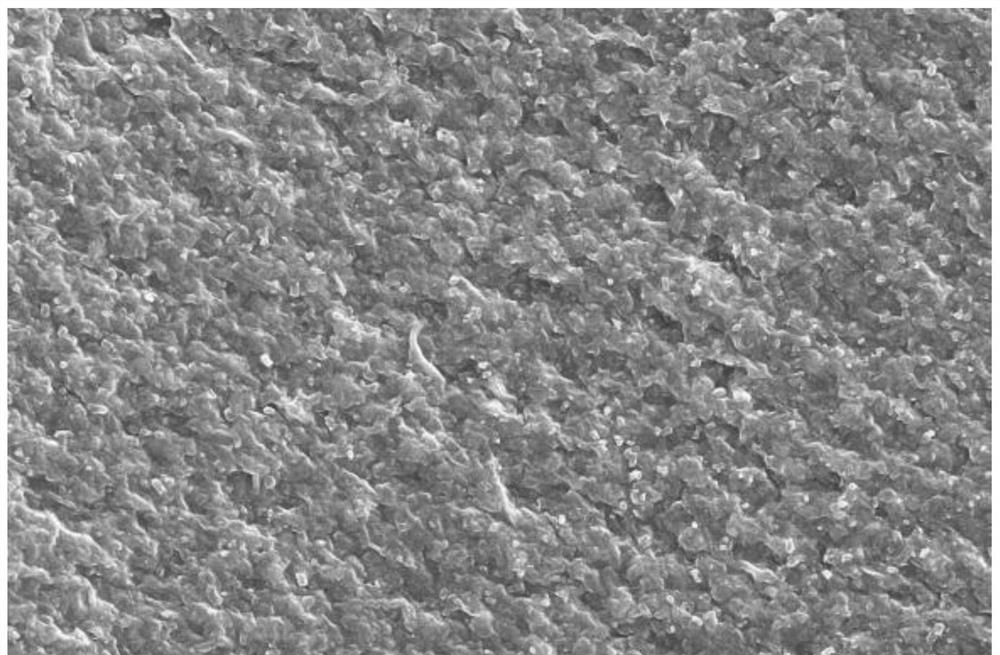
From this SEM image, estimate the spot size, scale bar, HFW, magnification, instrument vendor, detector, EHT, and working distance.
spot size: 10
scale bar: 30000 nm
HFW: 82.9 µm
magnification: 5 K X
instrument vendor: FEI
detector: ETD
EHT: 20 kV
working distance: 13.6 mm